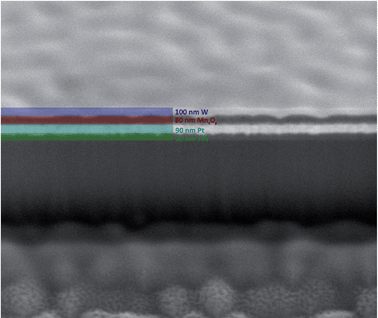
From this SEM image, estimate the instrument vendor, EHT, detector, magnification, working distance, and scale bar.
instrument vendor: FEI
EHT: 5 kV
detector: ETD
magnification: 100 K X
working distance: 5 mm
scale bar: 1000 nm